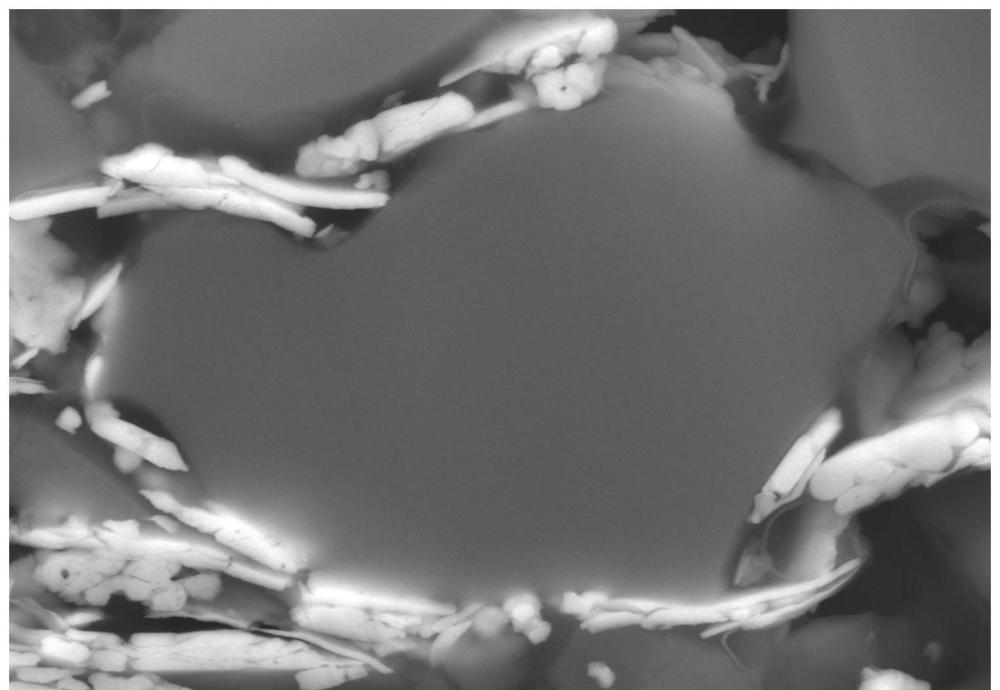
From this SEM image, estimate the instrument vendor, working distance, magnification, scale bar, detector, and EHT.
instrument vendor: Hitachi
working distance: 14.5 mm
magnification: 10 K X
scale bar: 5000 nm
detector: LA100(UL)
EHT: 20 kV